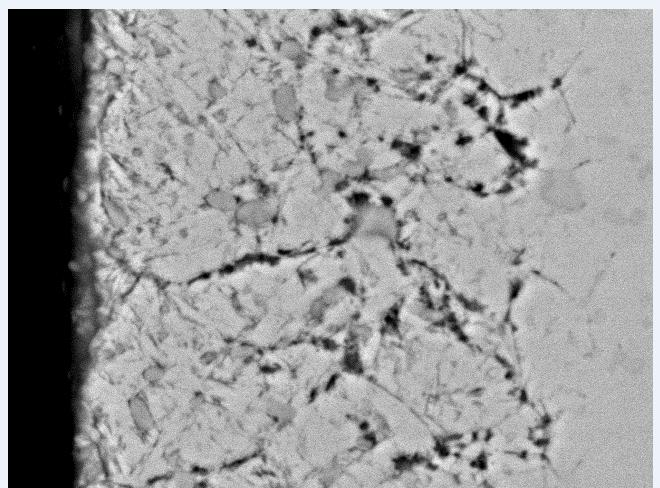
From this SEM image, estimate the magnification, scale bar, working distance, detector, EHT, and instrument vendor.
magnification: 8 K X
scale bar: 5000 nm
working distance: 10.2 mm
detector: BSE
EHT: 20 kV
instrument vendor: FEI